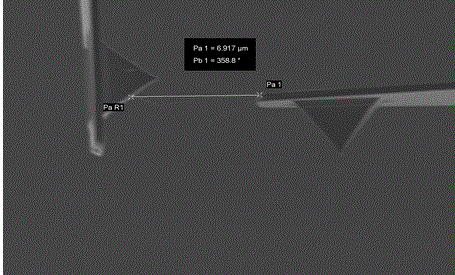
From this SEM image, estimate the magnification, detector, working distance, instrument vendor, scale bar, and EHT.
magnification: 4.7 K X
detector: SE2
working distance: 7.7 mm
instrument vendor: Zeiss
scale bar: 2000 nm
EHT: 5 kV